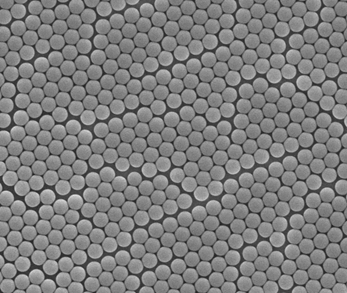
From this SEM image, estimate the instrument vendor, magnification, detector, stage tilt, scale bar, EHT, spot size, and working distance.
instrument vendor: FEI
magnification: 50 K X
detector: ETD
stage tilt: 0°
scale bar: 2000 nm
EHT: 10 kV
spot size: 3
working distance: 8.7 mm